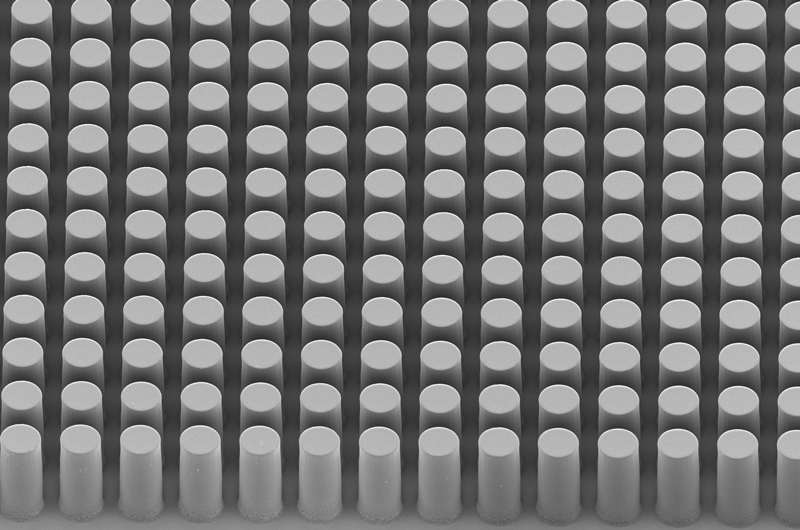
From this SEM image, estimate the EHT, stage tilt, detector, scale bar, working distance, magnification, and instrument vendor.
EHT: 3 kV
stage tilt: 45°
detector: InLens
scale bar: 20000 nm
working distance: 9.5 mm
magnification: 0.288 K X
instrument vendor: Zeiss